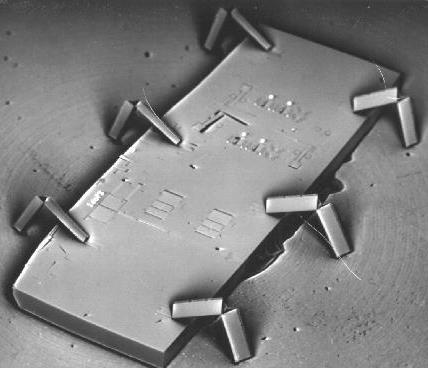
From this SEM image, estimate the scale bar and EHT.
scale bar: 1000000 nm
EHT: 20 kV